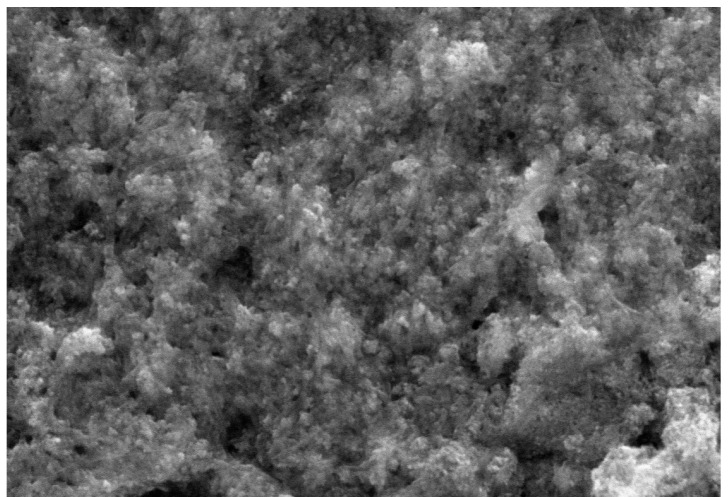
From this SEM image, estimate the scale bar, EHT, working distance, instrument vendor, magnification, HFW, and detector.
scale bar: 2000 nm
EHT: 10 kV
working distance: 15.03 mm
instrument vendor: Tescan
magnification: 71.8 K X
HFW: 9.64 µm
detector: SE